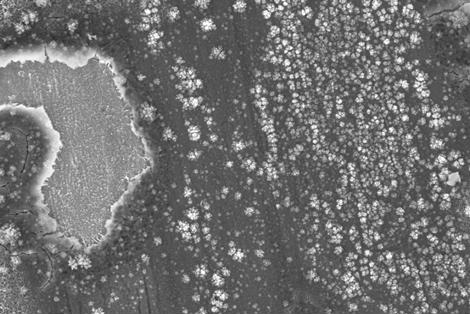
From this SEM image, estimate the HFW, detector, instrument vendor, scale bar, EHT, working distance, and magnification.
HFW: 19.86 µm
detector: InLens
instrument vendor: Zeiss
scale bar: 2000 nm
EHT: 5 kV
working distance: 4.3 mm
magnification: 15 K X